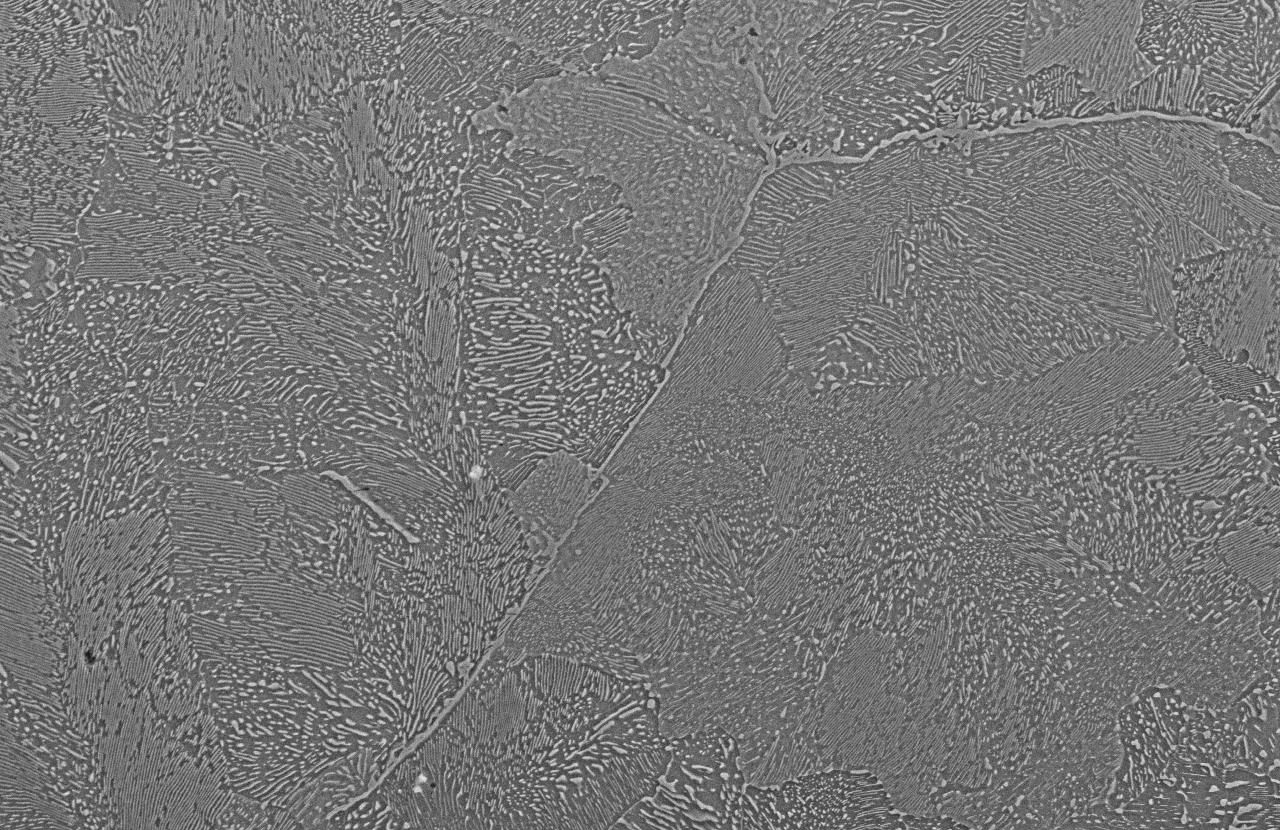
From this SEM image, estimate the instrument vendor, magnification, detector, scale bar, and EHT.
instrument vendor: JEOL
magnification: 1 K X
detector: SEI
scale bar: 20000 nm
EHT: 15 kV